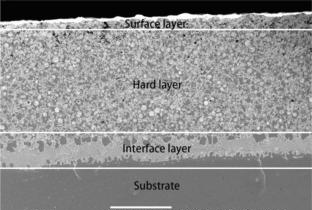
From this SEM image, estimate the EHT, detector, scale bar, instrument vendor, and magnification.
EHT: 20 kV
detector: SEI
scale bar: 500000 nm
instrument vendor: JEOL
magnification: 0.05 K X